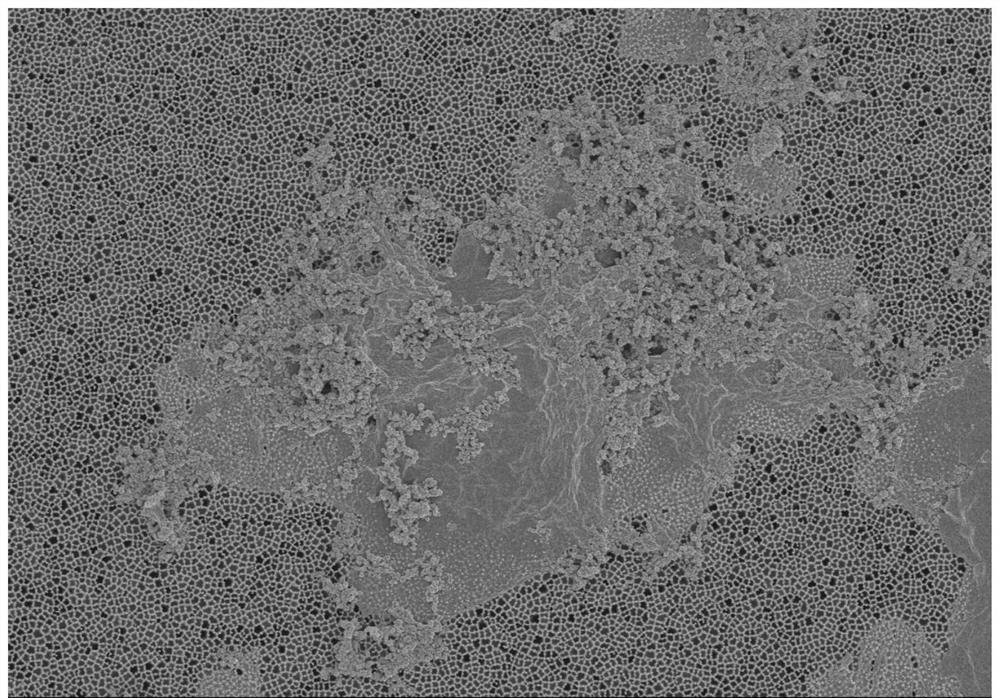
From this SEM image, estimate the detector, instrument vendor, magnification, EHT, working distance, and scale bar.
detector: SE(M)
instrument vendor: Hitachi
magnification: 6 K X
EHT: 3 kV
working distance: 8.3 mm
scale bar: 5000 nm